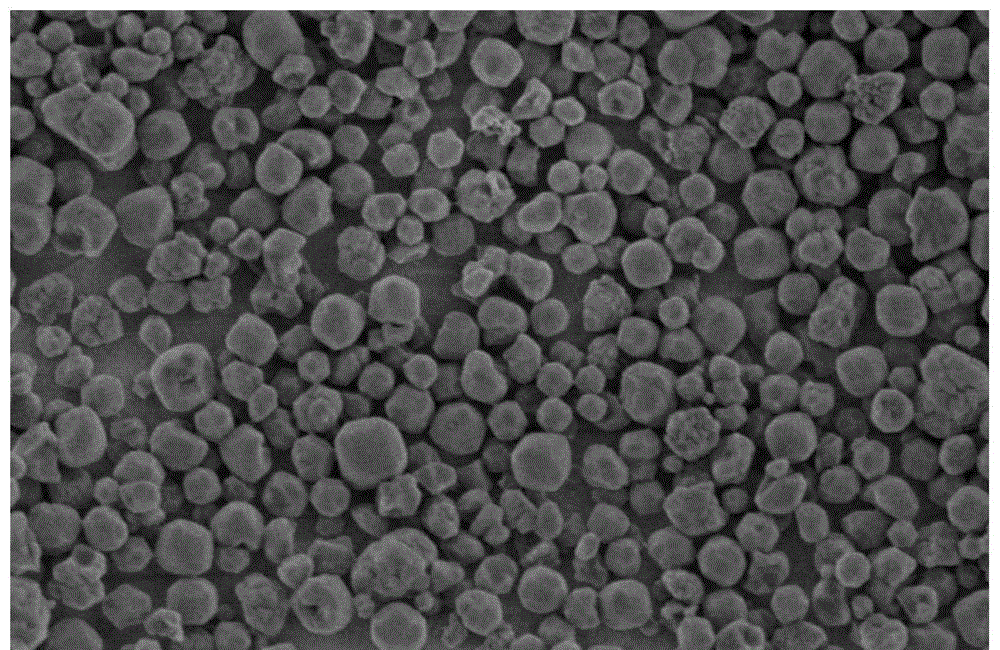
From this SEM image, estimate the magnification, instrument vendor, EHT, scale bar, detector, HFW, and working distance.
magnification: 50 K X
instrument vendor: Thermo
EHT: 0.7 kV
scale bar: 1000 nm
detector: TLD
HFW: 2.54 µm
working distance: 2 mm